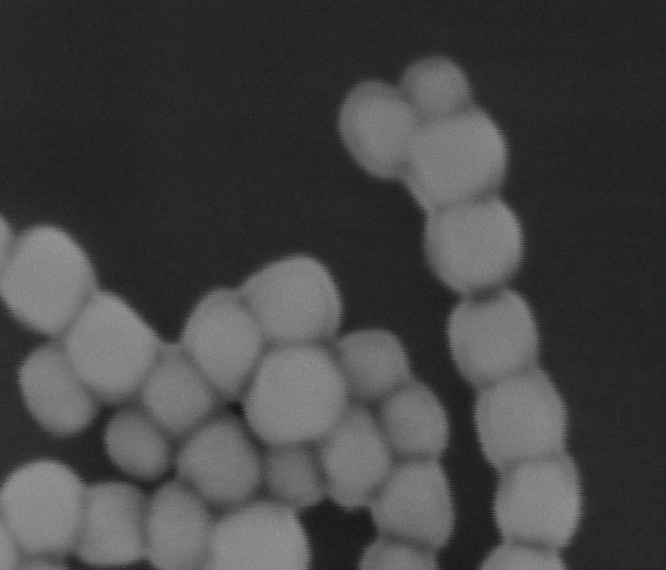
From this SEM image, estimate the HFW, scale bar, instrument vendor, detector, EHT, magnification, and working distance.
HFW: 0.432 µm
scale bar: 100 nm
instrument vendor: FEI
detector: TLD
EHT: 5 kV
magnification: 690.49 K X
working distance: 4.2 mm